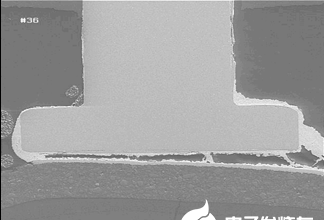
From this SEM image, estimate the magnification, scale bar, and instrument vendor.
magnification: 0.1 K X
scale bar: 200000 nm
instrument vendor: Hitachi